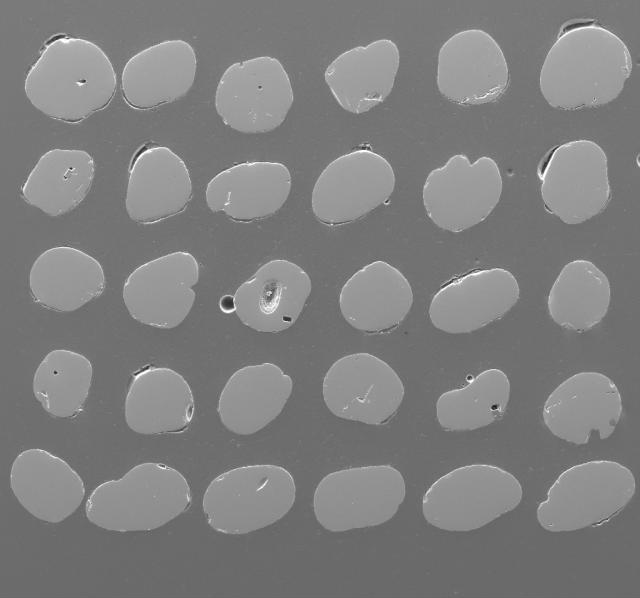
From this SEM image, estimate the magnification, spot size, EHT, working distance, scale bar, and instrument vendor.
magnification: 0.113 K X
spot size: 6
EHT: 15 kV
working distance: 18.2 mm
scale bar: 500000 nm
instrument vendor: FEI